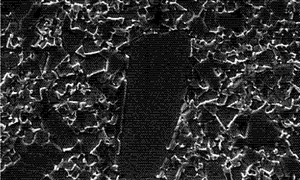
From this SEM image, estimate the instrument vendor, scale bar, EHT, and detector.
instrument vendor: Zeiss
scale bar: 2000 nm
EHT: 20 kV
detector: SE1